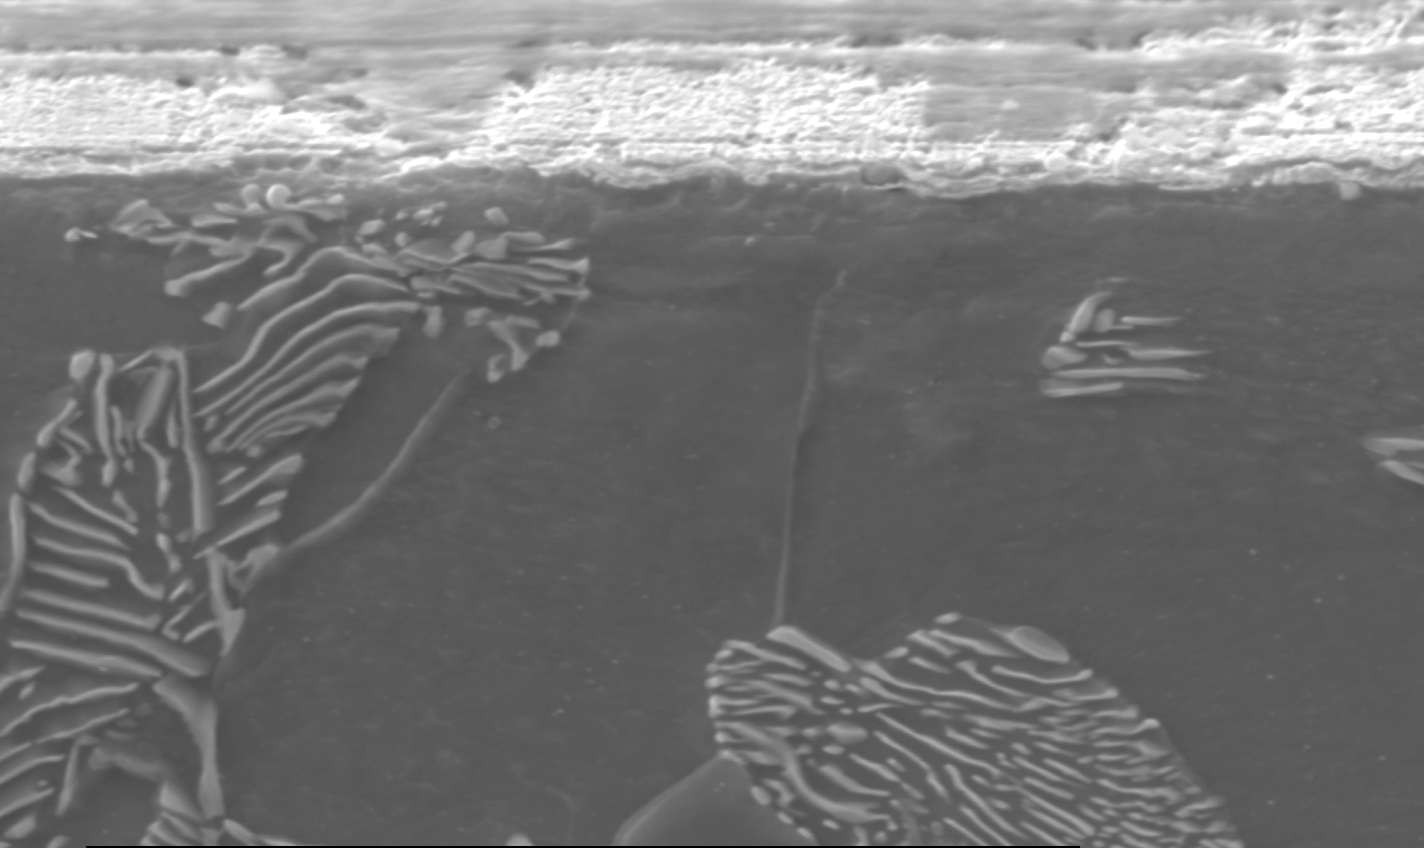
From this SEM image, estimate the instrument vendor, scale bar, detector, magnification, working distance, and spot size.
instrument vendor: FEI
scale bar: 10000 nm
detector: SE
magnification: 3.5 K X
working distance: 10.5 mm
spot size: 5